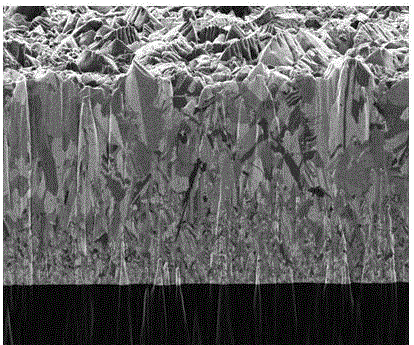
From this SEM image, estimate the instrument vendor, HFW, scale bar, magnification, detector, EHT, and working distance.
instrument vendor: FEI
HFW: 15.2 µm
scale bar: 2000 nm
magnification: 20 K X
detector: SED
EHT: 30 kV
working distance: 18 mm